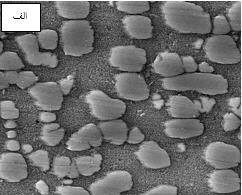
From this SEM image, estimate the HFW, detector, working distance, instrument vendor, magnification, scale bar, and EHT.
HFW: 5.93 µm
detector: SE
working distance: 13.18 mm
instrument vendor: Tescan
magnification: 35 K X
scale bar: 1000 nm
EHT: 15 kV